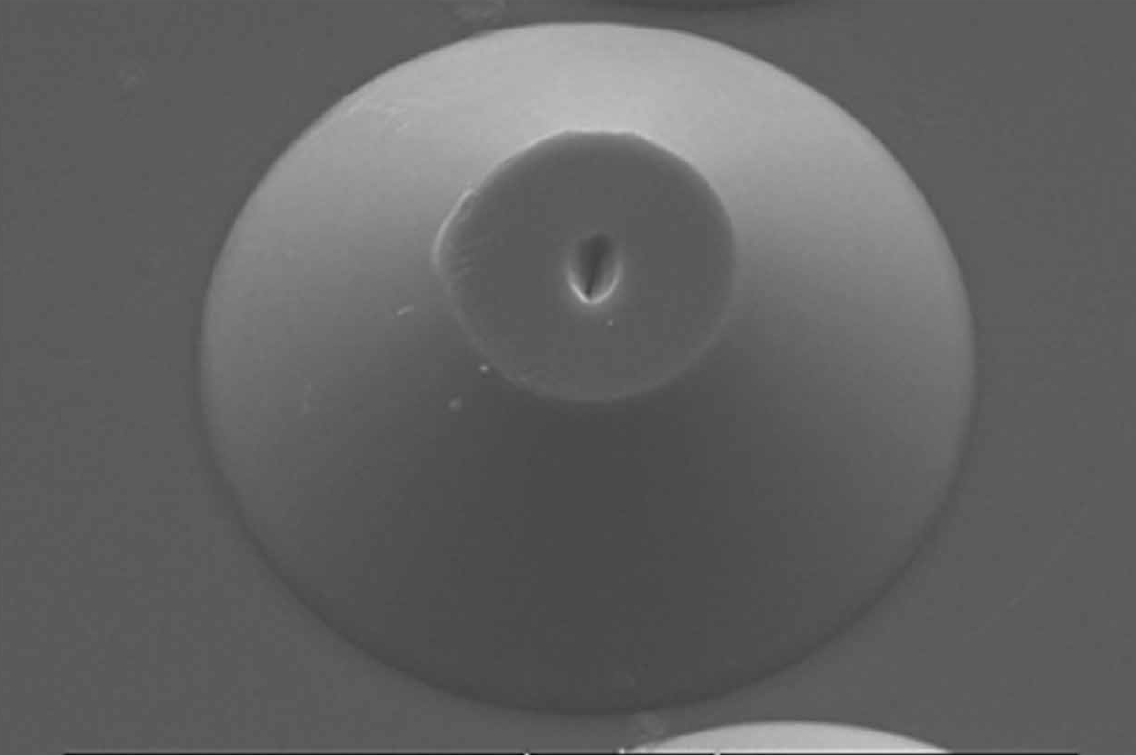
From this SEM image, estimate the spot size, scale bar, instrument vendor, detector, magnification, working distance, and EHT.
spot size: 4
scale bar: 20000 nm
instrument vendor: FEI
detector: SE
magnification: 1 K X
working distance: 9.5 mm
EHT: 15 kV